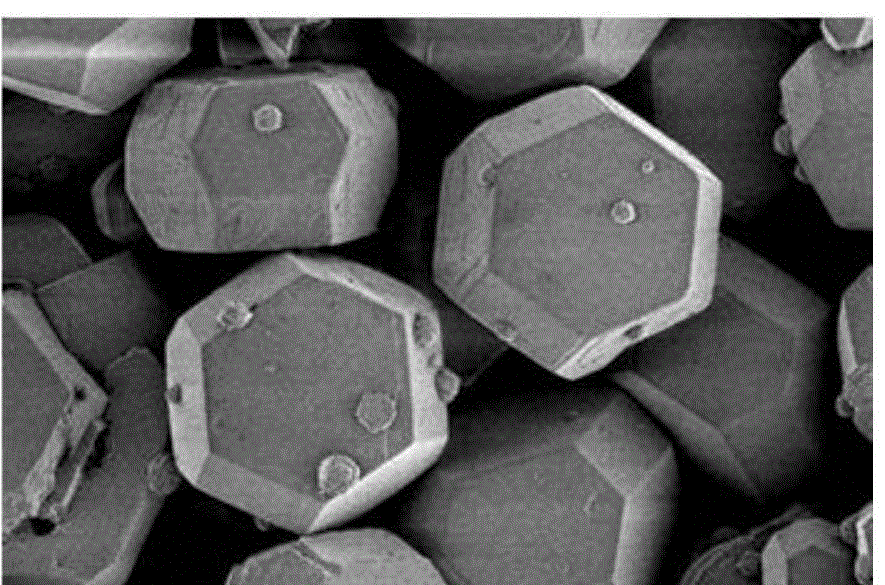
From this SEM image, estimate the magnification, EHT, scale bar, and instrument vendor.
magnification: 3 K X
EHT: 1 kV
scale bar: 10000 nm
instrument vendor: Hitachi